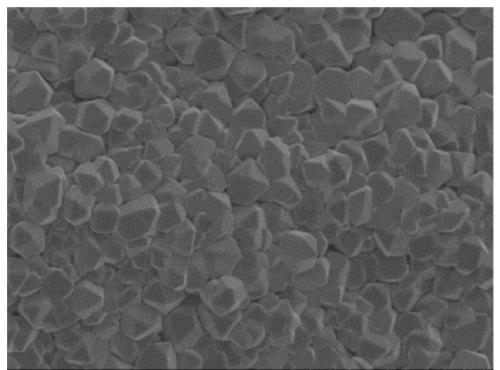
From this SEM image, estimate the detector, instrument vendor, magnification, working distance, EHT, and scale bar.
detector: SE(M)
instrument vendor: Hitachi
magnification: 5 K X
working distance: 9.3 mm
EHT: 3 kV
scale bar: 10000 nm